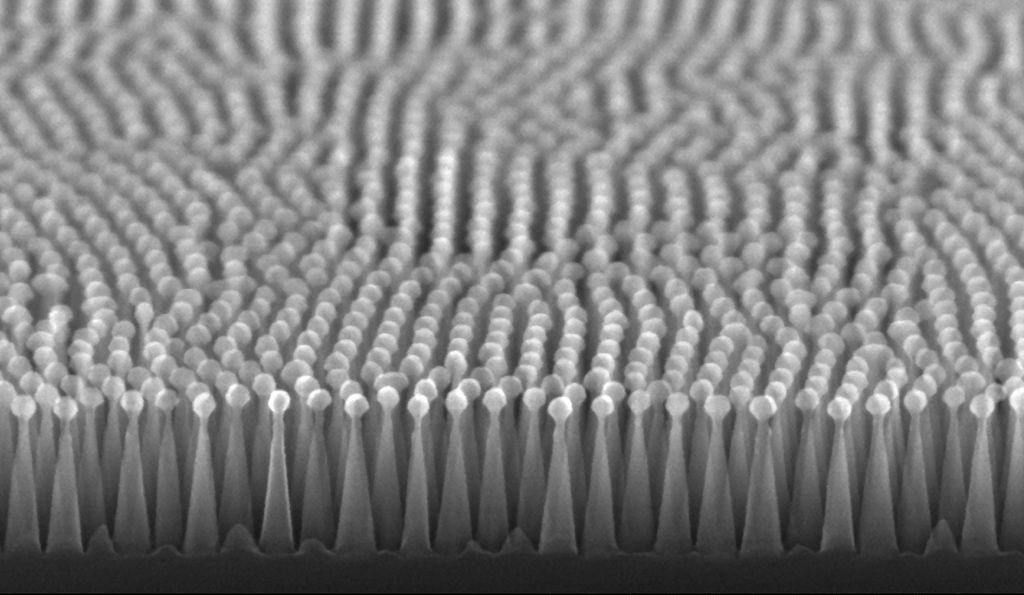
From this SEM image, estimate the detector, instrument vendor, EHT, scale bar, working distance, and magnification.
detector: SE(U,LA0)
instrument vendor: Hitachi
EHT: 20 kV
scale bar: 500 nm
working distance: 5.7 mm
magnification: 100 K X